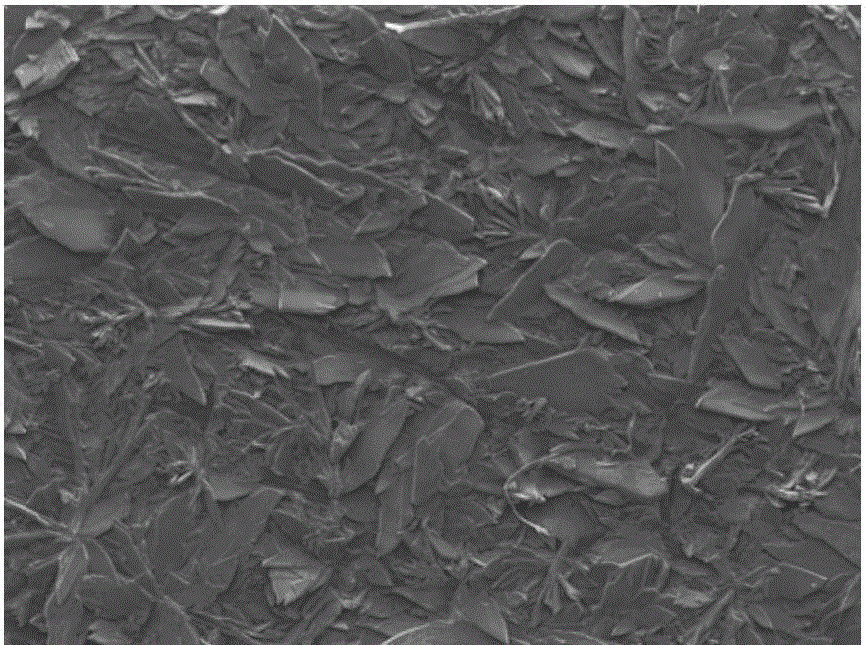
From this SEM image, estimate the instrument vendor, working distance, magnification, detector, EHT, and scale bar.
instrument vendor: JEOL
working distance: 14 mm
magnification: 0.05 K X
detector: SEI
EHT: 15 kV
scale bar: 100000 nm